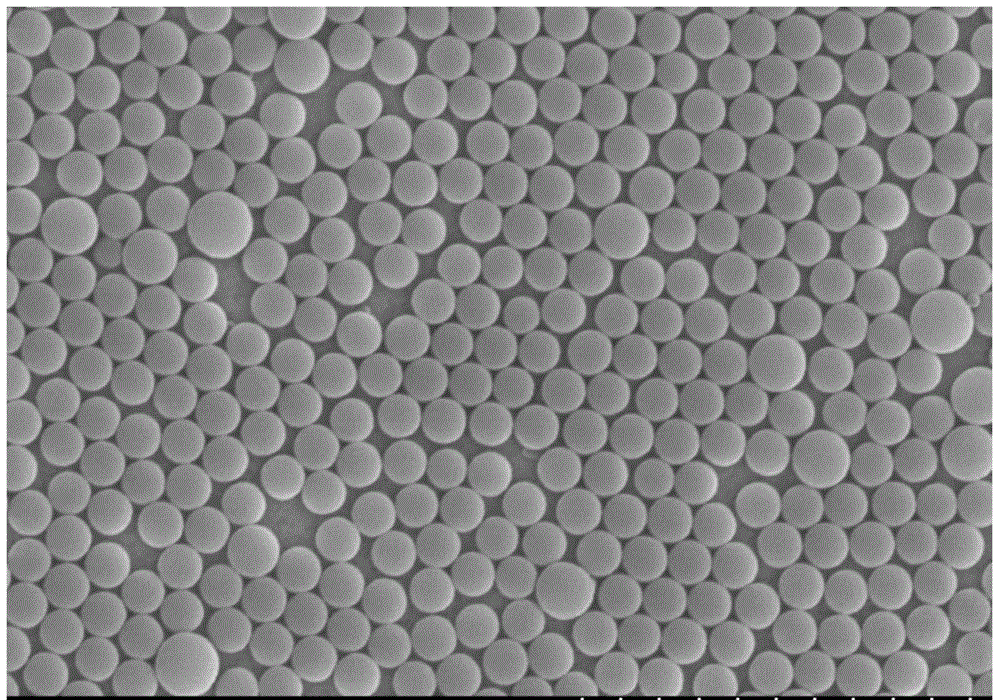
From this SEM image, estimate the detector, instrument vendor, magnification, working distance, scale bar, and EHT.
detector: SE(M,0)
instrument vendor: Hitachi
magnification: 10 K X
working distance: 13.3 mm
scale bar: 5000 nm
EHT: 15 kV